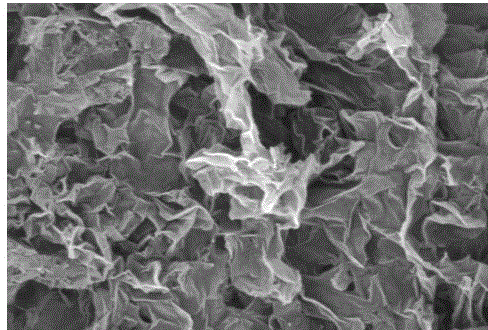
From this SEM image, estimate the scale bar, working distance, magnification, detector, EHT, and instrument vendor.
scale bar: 200 nm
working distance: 15.8 mm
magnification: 49.48 K X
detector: SE2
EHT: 3 kV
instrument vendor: Zeiss